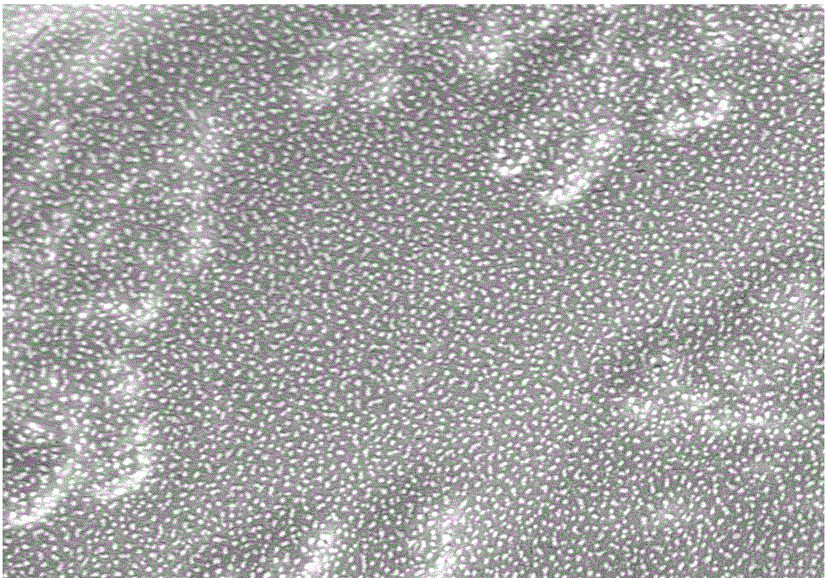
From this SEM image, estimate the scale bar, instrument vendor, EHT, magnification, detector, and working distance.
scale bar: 1000 nm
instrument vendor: Hitachi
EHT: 3 kV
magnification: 50 K X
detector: SE(M)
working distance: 8.5 mm